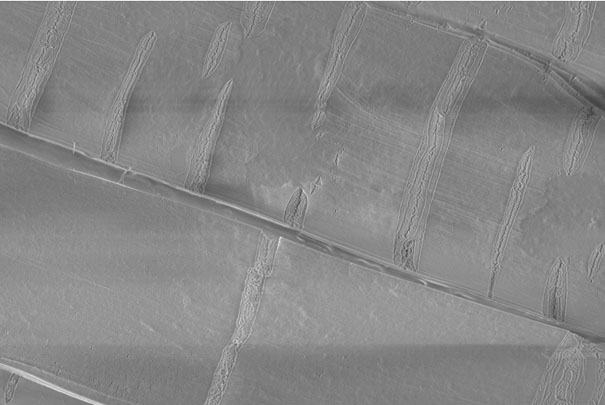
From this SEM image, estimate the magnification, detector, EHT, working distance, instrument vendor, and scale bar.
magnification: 2 K X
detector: InLens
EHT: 2 kV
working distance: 4.6 mm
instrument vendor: Zeiss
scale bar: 2000 nm